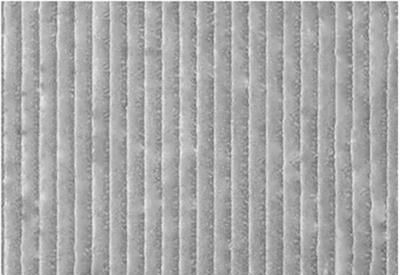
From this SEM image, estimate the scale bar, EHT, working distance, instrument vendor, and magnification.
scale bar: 5000 nm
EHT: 5 kV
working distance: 6.6 mm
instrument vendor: Hitachi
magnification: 10 K X